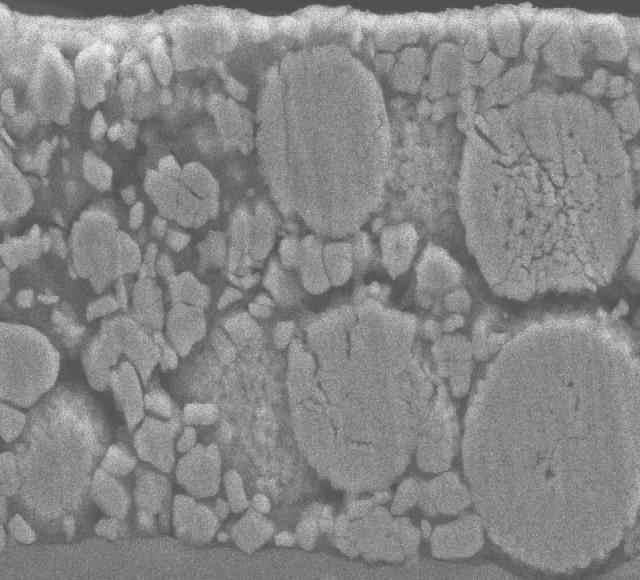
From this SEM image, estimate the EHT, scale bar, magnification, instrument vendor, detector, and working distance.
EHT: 15 kV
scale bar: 5000 nm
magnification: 3 K X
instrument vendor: JEOL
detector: SEI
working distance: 8 mm